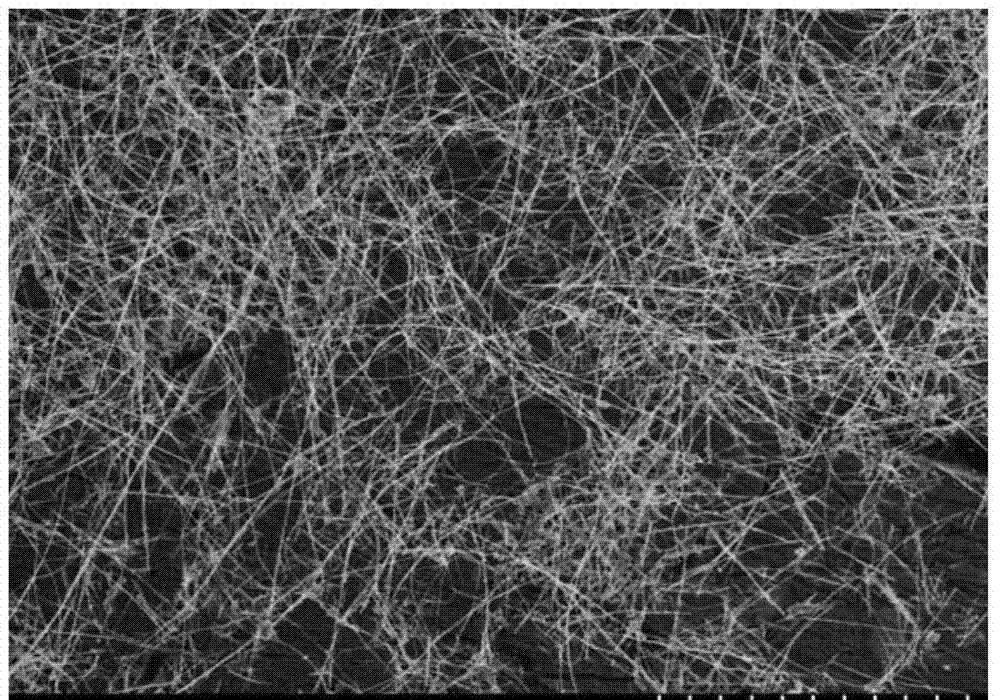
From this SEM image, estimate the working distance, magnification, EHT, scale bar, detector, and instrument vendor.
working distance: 8.2 mm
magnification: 2 K X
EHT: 5 kV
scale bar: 20000 nm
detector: SE(M)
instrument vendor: Hitachi